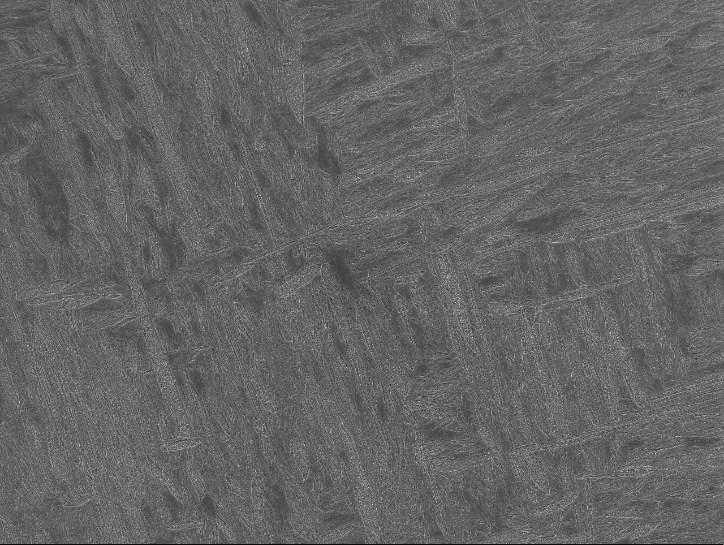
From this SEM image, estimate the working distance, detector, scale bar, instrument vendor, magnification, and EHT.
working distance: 9.8 mm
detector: SEI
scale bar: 10000 nm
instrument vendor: JEOL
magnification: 1 K X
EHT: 15 kV